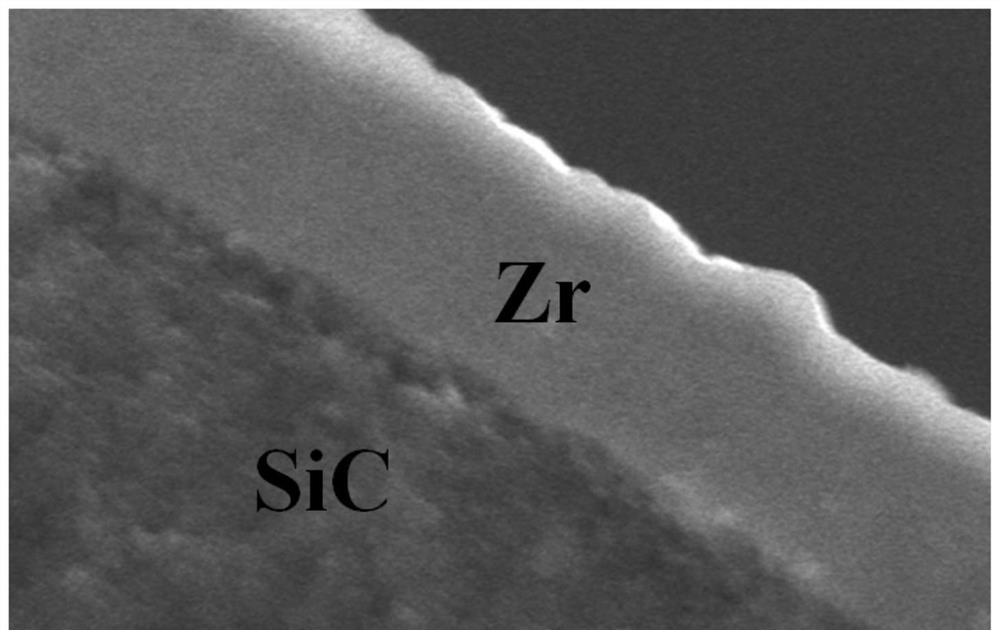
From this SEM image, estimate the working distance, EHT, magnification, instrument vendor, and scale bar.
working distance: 15 mm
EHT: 20 kV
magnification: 2.3 K X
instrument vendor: Hitachi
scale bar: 20000 nm